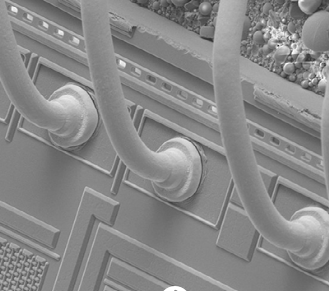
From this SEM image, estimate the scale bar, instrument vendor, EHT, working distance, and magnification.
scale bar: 100000 nm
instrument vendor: FEI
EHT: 10 kV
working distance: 5.8 mm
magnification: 1.2 K X